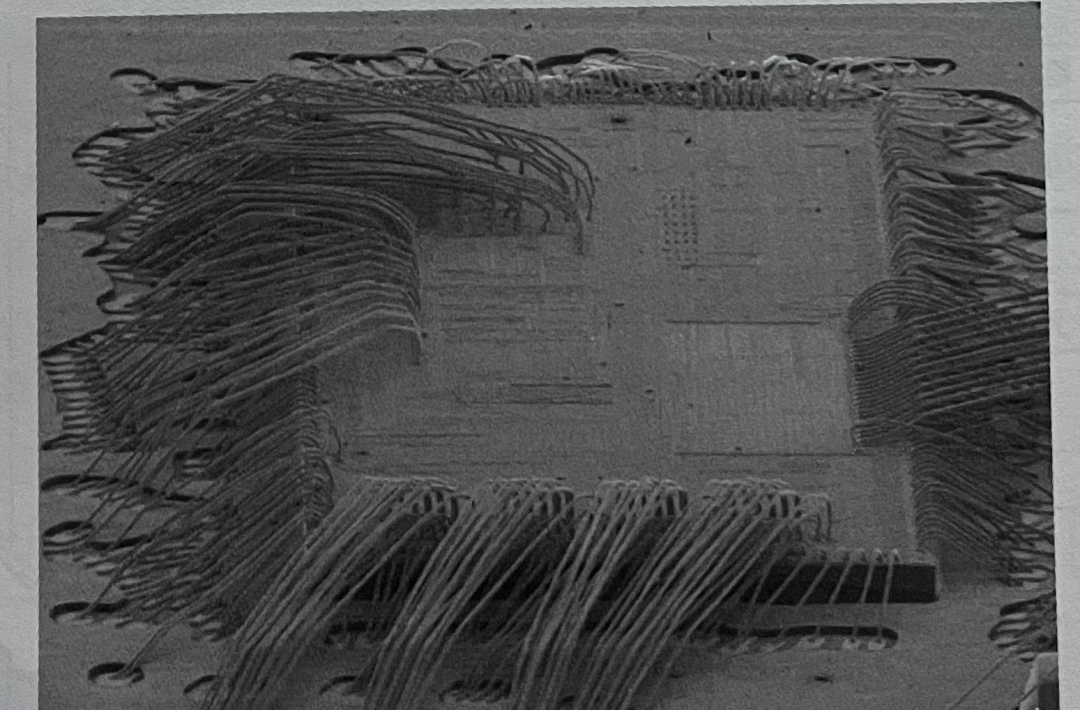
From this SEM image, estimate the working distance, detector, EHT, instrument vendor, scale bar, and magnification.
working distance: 8 mm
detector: LM(UL)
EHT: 10 kV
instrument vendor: Hitachi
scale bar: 1000000 nm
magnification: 0.03 K X